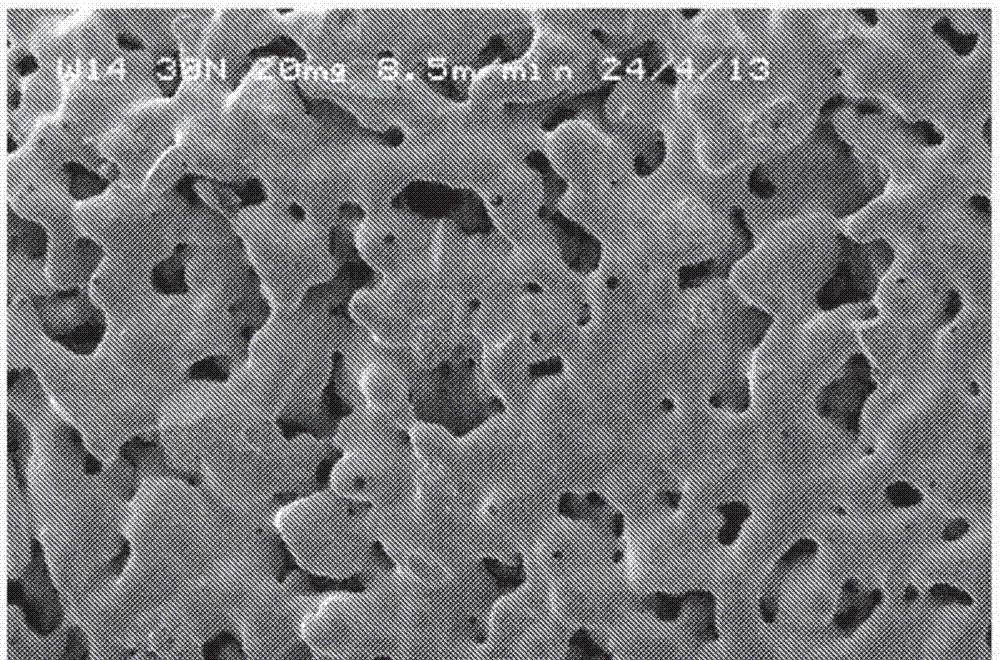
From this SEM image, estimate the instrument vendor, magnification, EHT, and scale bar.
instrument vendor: JEOL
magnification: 0.07 K X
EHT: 5 kV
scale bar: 200000 nm